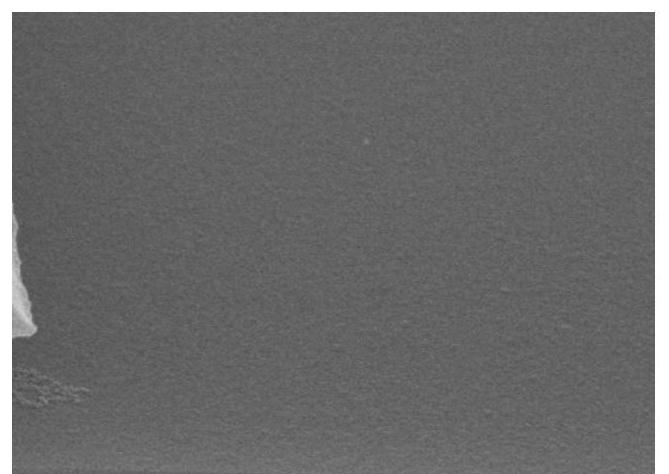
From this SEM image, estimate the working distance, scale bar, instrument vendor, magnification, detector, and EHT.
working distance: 11.8 mm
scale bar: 1000 nm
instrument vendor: Hitachi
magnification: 50 K X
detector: SE(U)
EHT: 10 kV